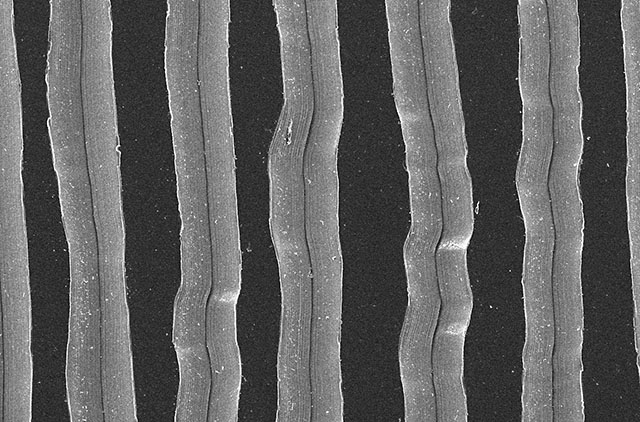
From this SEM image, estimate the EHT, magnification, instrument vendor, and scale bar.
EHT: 10 kV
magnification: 0.15 K X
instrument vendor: JEOL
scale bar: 100000 nm